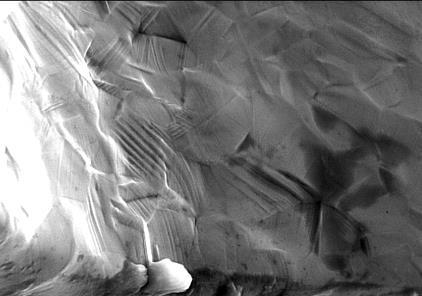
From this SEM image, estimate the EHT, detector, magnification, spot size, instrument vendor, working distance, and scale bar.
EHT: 25 kV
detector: SE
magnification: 2.522 K X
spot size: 4.7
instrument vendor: FEI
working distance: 16.5 mm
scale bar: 20000 nm